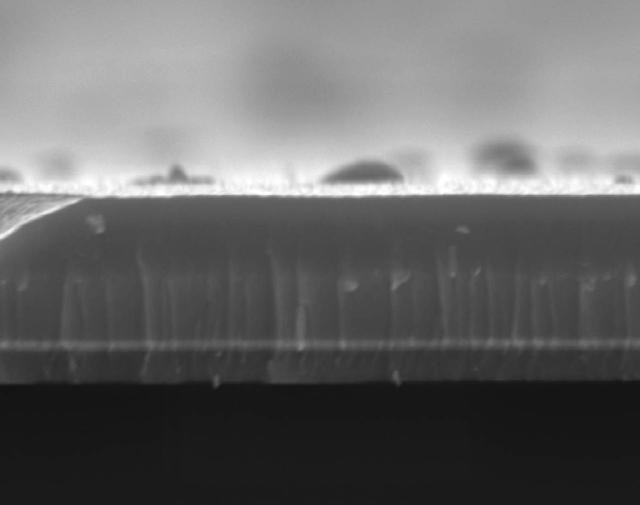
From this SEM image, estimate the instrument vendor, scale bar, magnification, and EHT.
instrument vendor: JEOL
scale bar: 1000 nm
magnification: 10 K X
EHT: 20 kV